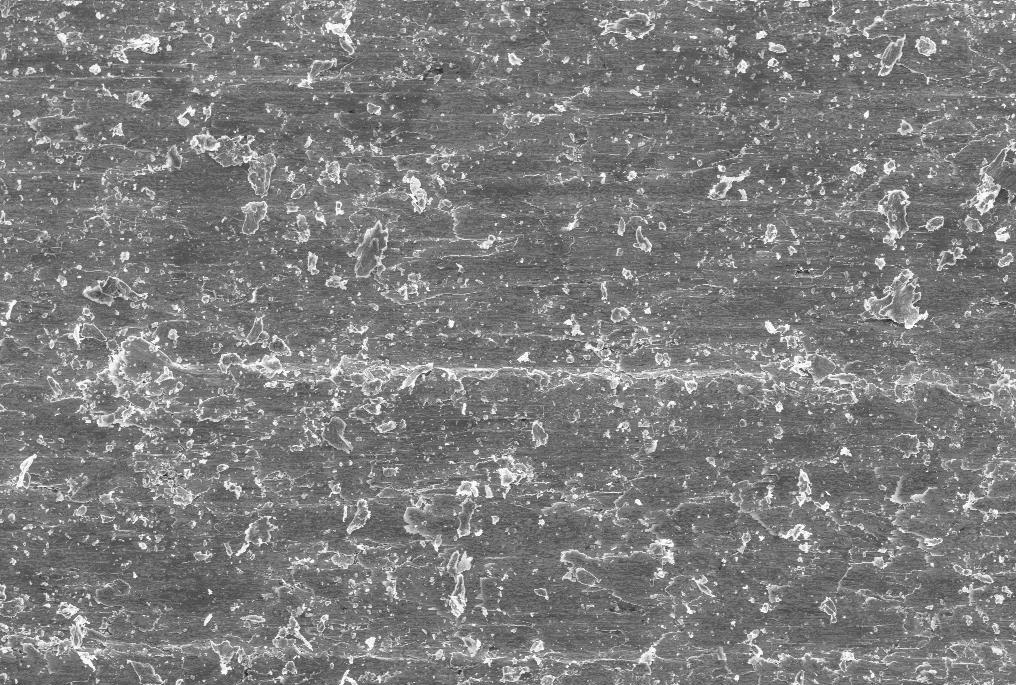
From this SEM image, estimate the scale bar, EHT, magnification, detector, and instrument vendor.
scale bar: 100000 nm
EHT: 20 kV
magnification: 0.1 K X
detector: SE1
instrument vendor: Zeiss